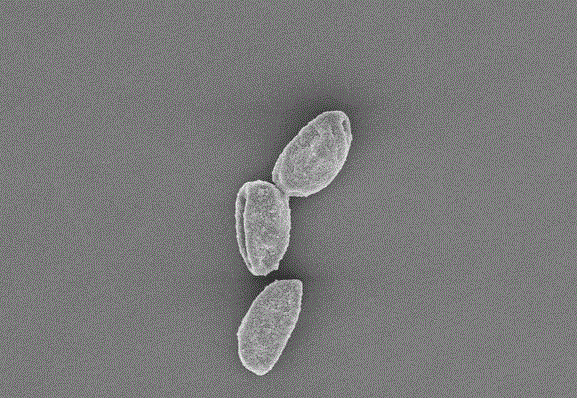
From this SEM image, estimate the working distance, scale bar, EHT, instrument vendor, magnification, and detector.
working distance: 9.2 mm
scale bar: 10000 nm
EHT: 5 kV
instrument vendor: Hitachi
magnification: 3 K X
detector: SE(U)